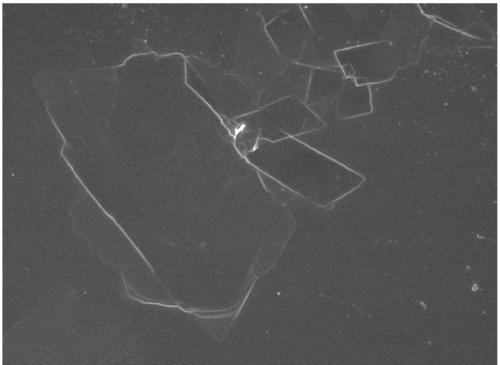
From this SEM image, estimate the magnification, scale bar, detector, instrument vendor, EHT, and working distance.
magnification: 2.7 K X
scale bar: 10000 nm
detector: SEI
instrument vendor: JEOL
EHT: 5 kV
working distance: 8.4 mm